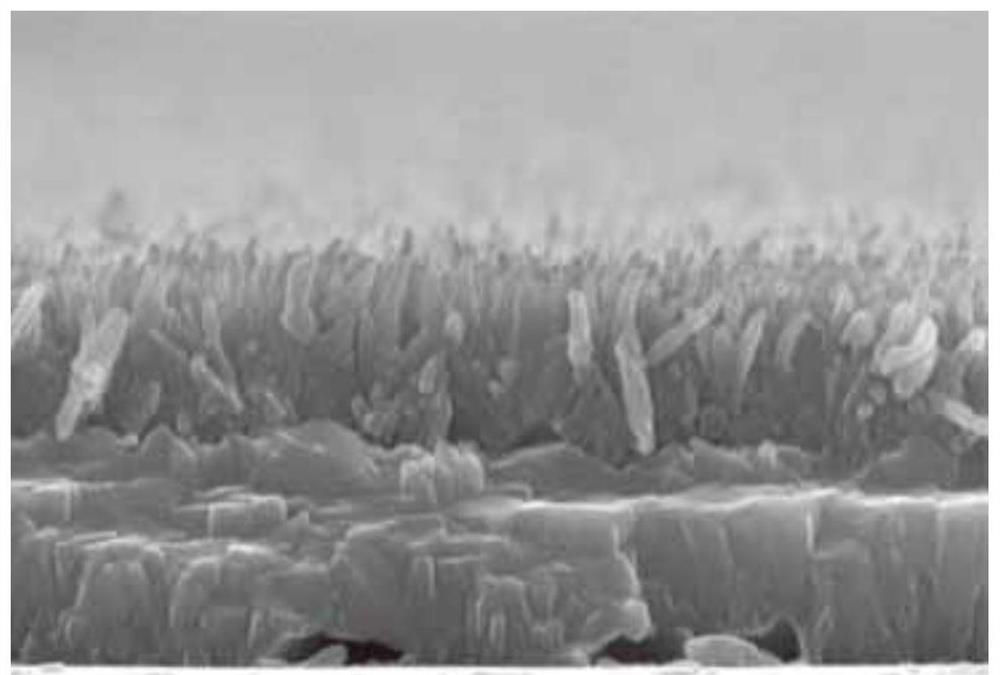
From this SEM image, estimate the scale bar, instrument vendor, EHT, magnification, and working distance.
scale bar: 1000 nm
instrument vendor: Hitachi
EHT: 15 kV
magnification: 50 K X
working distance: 11.2 mm